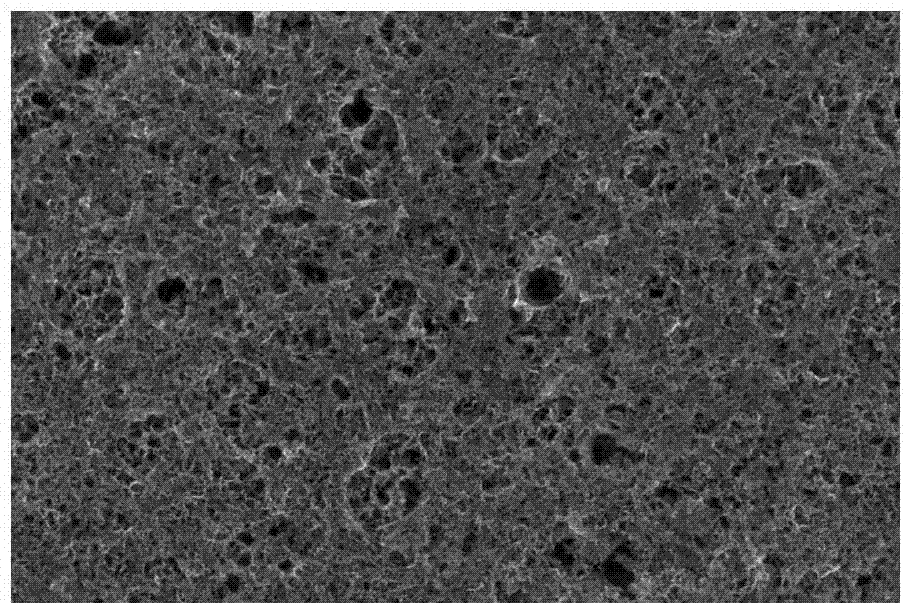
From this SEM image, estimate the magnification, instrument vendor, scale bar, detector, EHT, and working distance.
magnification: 200 K X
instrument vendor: FEI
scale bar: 500 nm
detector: TLD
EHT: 15 kV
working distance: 5.6 mm